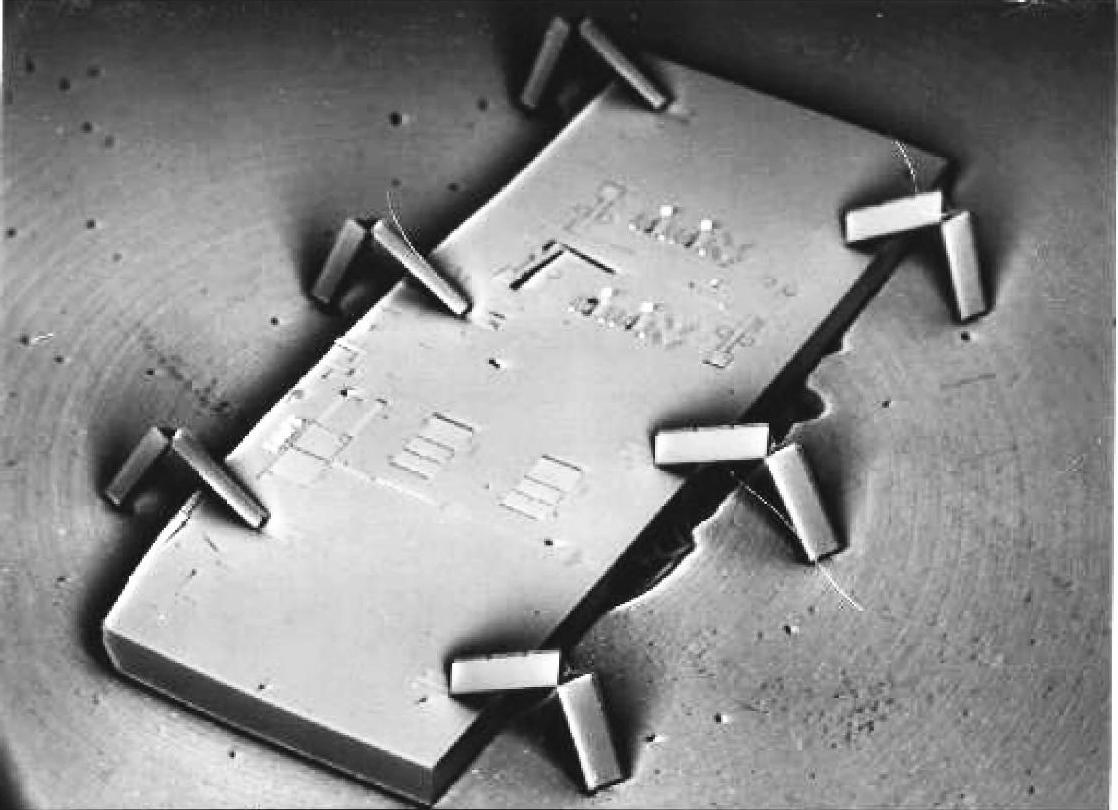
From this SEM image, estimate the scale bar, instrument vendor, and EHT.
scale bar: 1000000 nm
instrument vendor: other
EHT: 20 kV